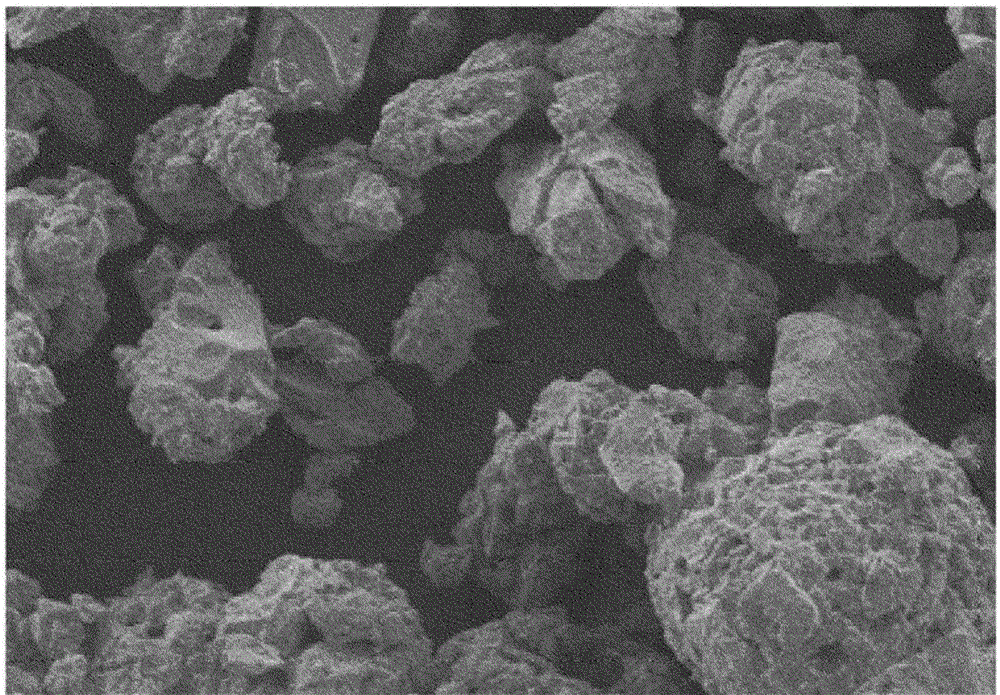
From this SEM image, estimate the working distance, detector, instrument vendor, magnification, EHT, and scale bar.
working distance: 16.2 mm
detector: SE(M)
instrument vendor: Hitachi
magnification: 0.5 K X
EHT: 5 kV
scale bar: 100000 nm